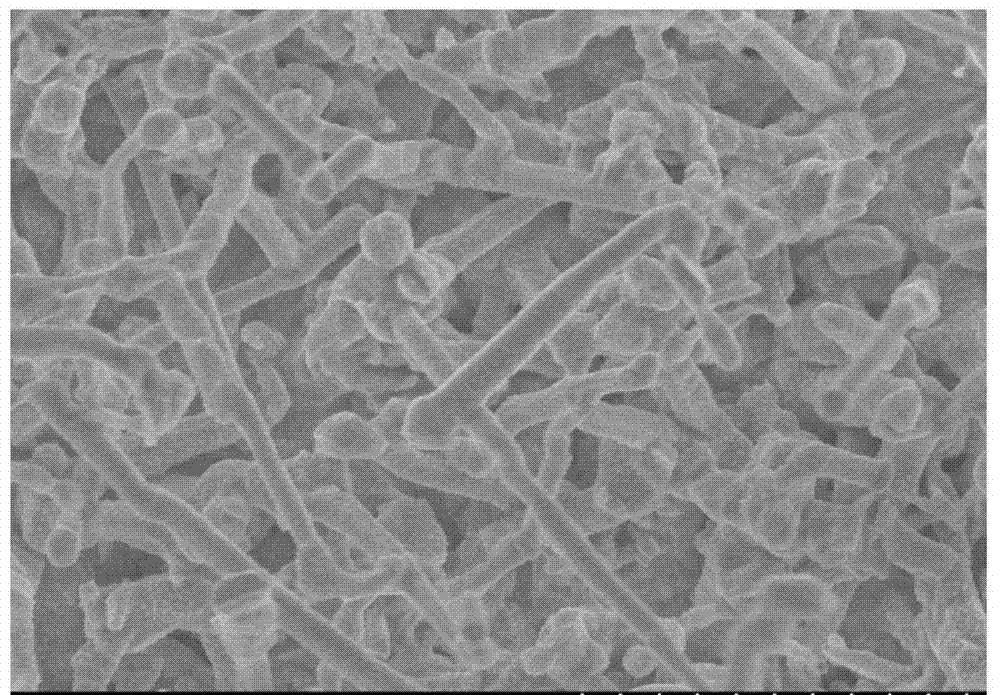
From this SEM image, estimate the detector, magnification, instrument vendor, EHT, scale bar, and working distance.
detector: SE(M)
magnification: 10 K X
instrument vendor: Hitachi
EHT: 5 kV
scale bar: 5000 nm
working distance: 6.7 mm